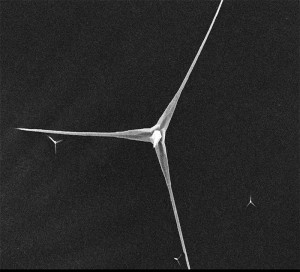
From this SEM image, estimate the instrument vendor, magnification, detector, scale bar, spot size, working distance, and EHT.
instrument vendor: other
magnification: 1.5 K X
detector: SE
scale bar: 10000 nm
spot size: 6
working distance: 8.6 mm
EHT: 15 kV